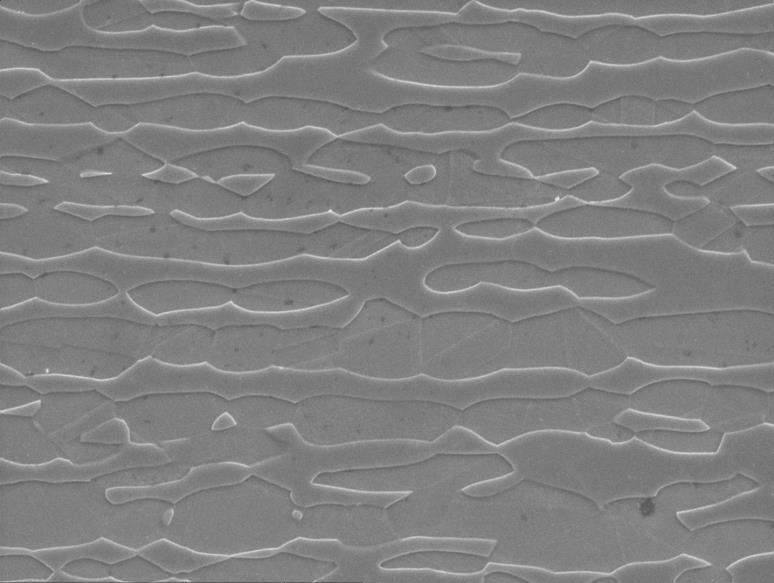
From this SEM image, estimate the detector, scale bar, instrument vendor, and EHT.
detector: SEI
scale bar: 10000 nm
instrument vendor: JEOL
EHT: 20 kV